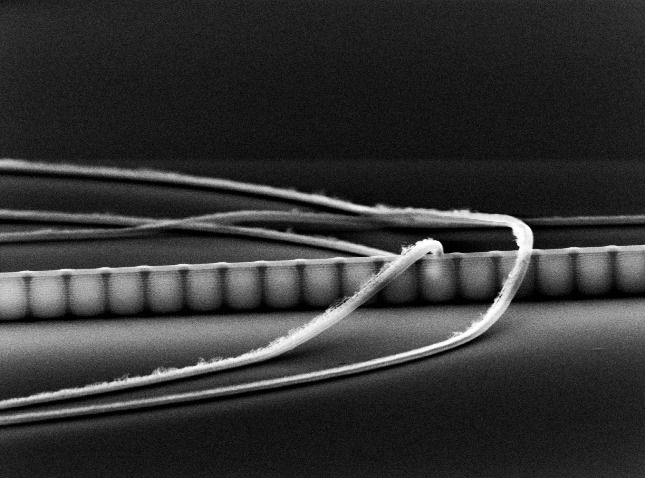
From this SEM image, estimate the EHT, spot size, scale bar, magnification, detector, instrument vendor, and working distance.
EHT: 30 kV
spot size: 4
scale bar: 2000 nm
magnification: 15.172 K X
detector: SE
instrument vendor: FEI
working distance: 13.1 mm